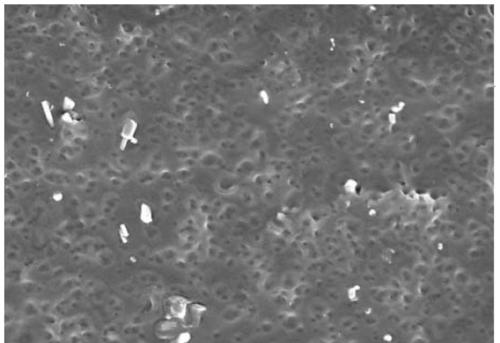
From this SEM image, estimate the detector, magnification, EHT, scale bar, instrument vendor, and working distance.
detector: SE(M)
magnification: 10 K X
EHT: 5 kV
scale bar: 5000 nm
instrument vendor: Hitachi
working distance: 8 mm